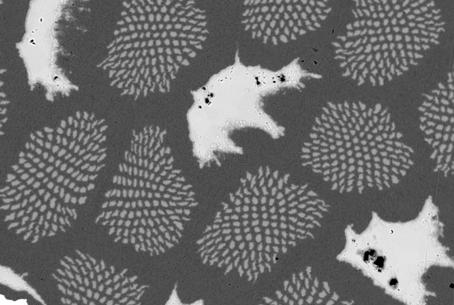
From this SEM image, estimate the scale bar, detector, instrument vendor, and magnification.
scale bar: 10000 nm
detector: QBSD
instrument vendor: Zeiss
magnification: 1.13 K X